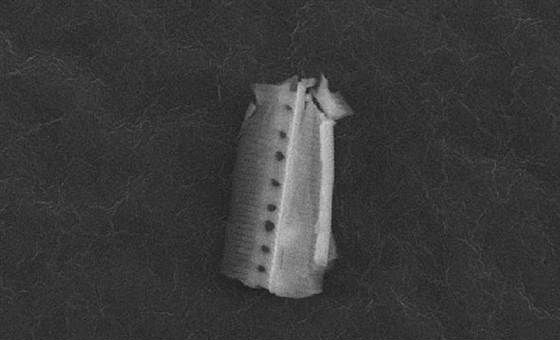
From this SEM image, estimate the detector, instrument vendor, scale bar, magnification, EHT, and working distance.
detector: SEI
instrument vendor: JEOL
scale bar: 1000 nm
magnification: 3.5 K X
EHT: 15 kV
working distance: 23 mm